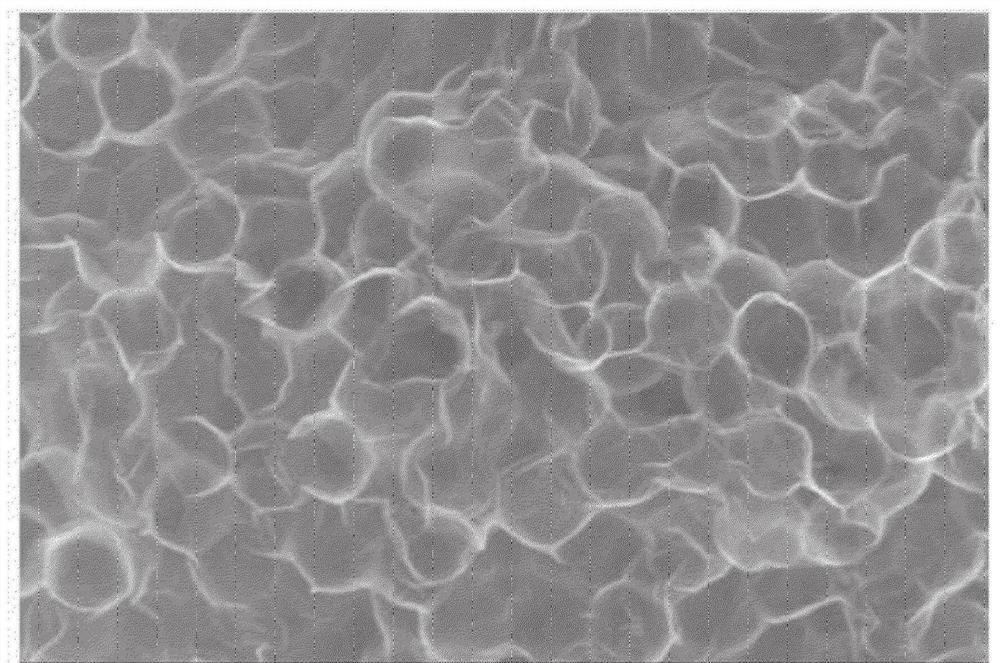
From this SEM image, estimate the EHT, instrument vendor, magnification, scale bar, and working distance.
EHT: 5 kV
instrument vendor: Hitachi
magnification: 50 K X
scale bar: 1000 nm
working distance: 10.2 mm